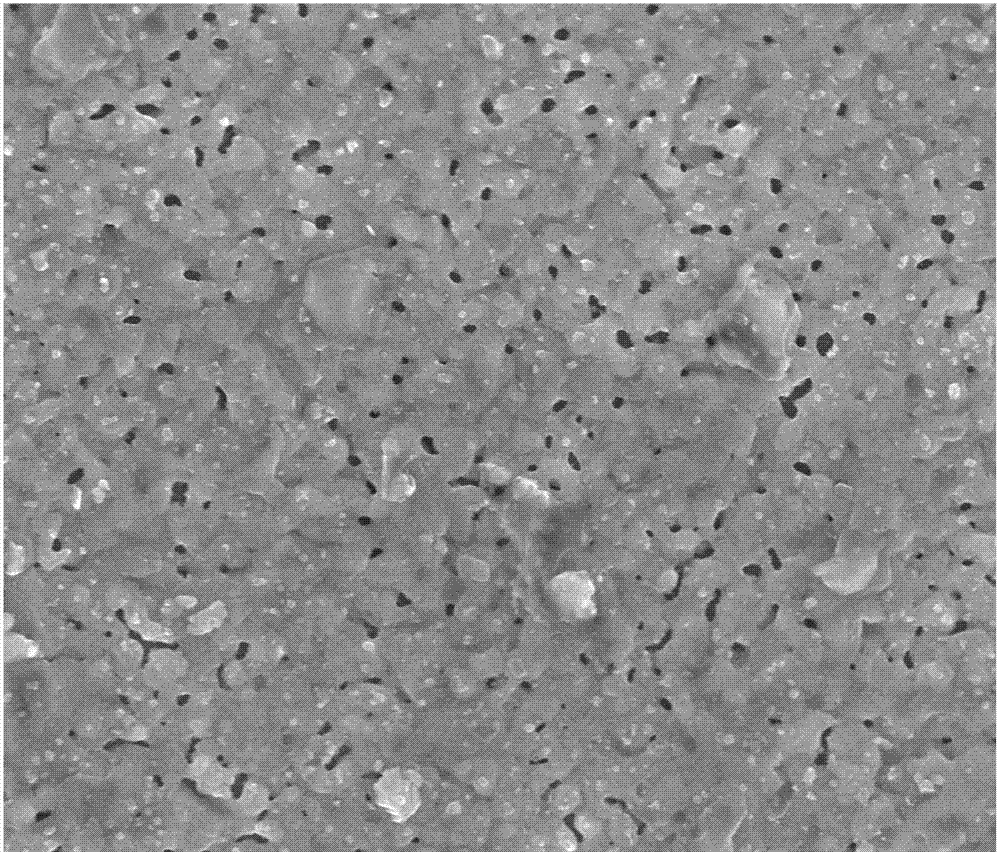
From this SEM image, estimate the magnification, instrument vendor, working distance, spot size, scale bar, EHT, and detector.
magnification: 20 K X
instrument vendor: FEI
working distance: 6.4 mm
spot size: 4.5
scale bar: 3000 nm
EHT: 18 kV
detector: TLD